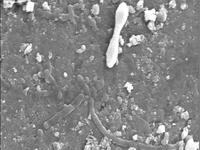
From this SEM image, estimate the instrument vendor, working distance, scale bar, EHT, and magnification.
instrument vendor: JEOL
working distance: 12 mm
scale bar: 1000 nm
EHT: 9 kV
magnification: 7.5 K X